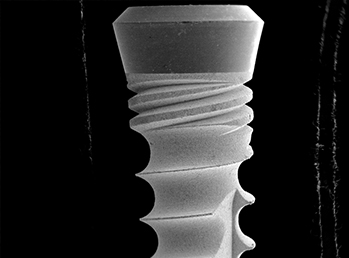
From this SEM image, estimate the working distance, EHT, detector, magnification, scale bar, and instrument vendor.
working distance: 42 mm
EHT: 15 kV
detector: SE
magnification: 0.017 K X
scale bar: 3000000 nm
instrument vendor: other